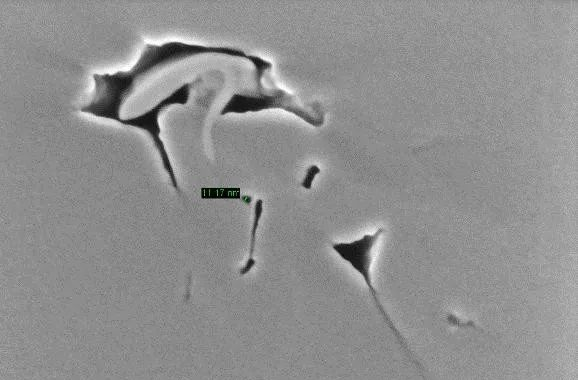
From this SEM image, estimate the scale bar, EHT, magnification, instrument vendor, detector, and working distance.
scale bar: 100 nm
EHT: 1 kV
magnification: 100 K X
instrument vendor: Zeiss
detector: InLens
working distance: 1.7 mm